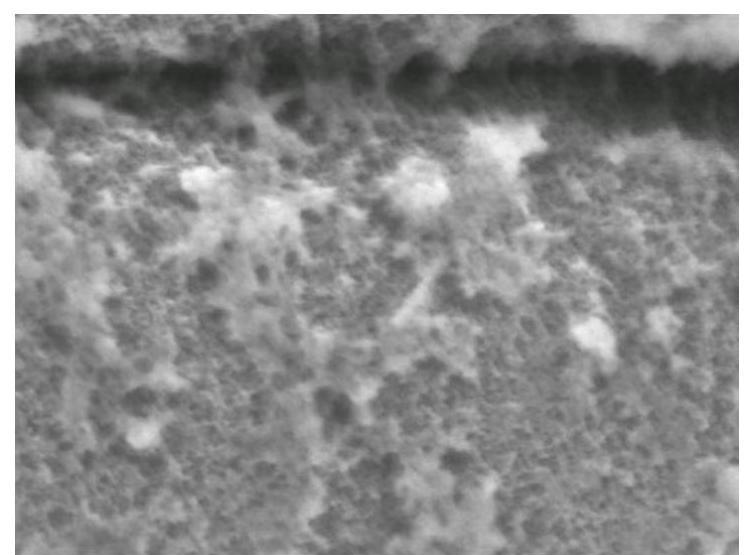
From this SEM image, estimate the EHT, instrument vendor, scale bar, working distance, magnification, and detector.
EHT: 5 kV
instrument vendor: JEOL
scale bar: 100 nm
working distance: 8 mm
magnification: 100 K X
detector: SEI